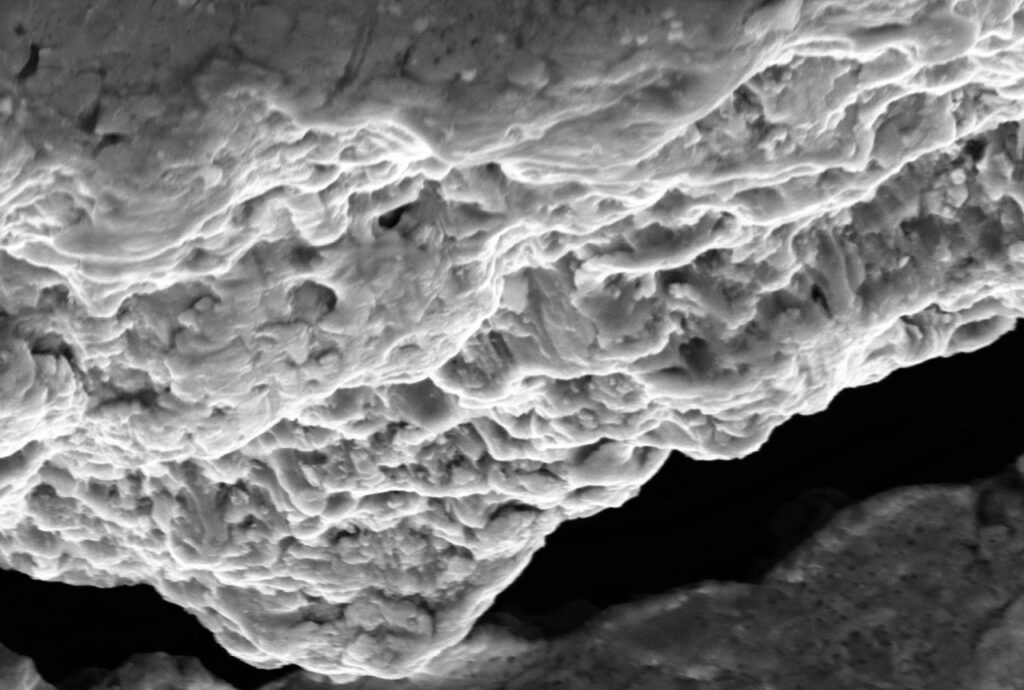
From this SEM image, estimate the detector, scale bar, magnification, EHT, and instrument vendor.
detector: SED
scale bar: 20000 nm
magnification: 1.3 K X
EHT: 15 kV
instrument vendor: JEOL